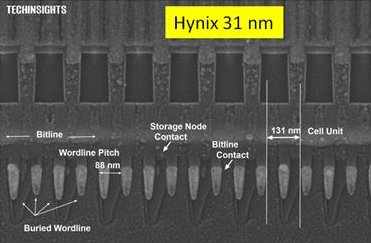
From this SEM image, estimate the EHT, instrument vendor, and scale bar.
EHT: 5 kV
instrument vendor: Zeiss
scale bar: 100 nm